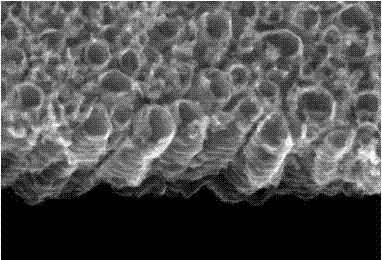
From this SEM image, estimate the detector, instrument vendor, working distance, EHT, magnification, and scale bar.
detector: SE(M)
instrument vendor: Hitachi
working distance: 10.6 mm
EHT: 15 kV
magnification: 100 K X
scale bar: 500 nm